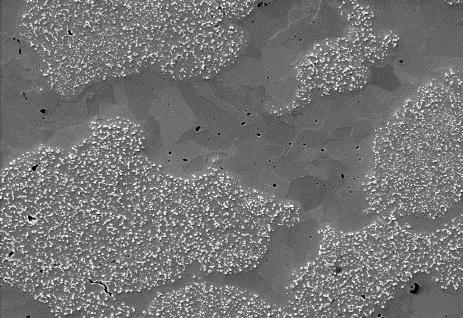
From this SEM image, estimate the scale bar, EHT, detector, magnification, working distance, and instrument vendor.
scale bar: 100000 nm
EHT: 20 kV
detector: SE(M)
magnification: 0.5 K X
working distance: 14.9 mm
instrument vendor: Hitachi